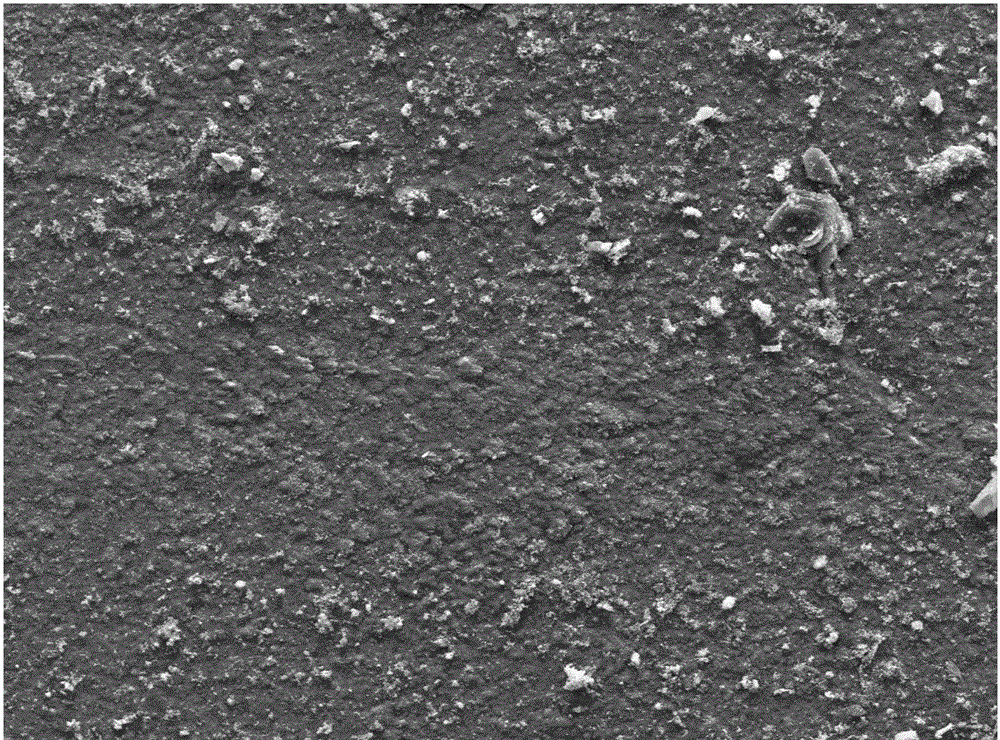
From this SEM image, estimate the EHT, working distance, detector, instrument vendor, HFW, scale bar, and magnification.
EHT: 30 kV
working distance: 9.3 mm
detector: ETD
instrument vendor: FEI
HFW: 512 µm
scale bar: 200000 nm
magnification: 0.5 K X